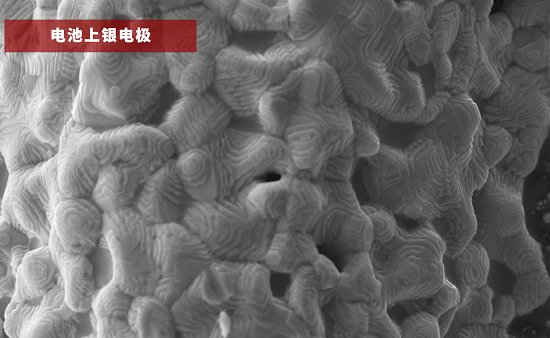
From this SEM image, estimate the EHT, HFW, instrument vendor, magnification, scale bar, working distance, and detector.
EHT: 15 kV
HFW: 25.4 µm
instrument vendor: Thermo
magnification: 5 K X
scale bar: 8000 nm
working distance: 4.827 mm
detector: SED